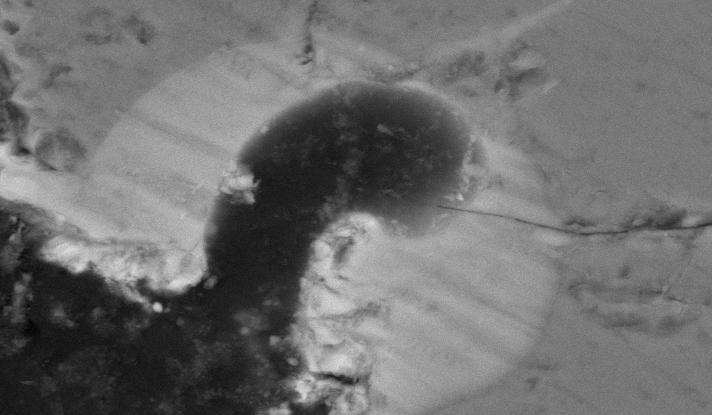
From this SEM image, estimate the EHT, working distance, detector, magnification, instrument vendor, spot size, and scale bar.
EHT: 20 kV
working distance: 10.1 mm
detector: BSE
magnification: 2 K X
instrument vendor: FEI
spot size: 5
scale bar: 10000 nm